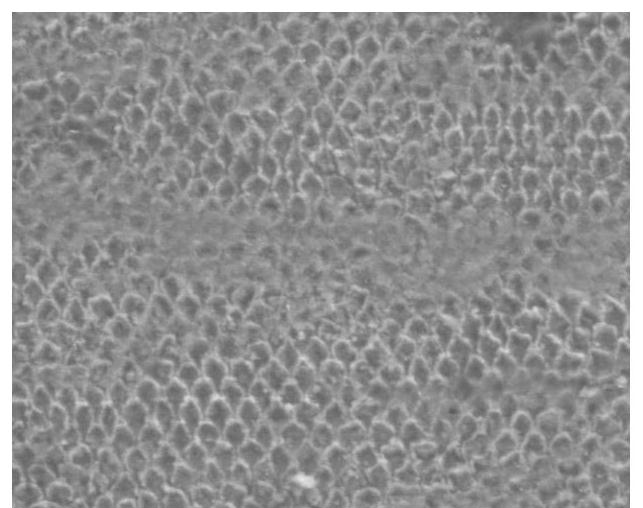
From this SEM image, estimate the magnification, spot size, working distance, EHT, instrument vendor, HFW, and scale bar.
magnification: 2 K X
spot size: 3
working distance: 10.6 mm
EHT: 15 kV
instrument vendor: FEI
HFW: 150 µm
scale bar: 50000 nm